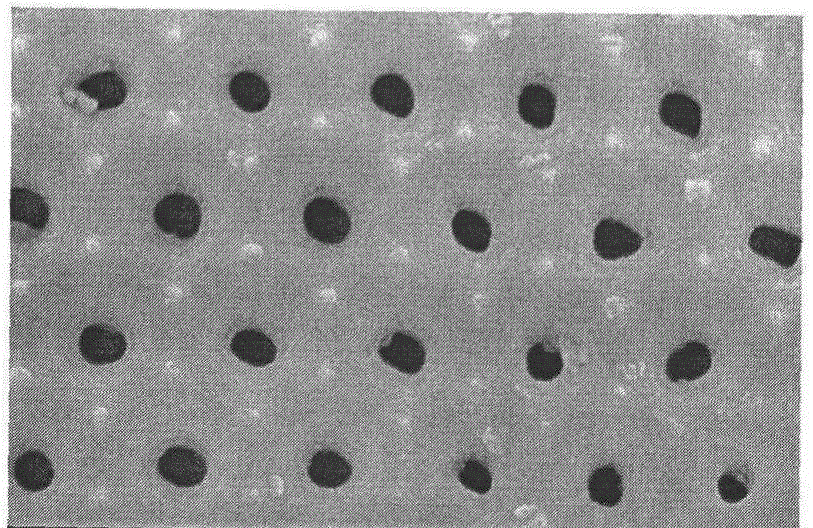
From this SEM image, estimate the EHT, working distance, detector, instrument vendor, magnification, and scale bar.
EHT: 10 kV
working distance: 6.9 mm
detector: SE(M)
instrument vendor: Hitachi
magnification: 50 K X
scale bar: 1000 nm